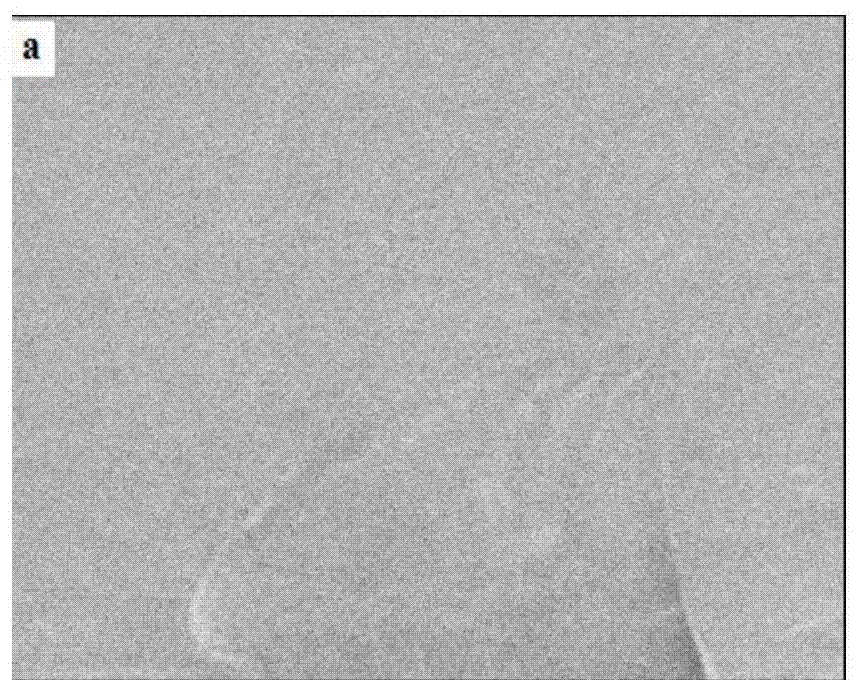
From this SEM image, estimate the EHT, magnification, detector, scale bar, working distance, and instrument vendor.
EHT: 5 kV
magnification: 0.8 K X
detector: SE(U)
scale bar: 50000 nm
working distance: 9 mm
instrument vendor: Hitachi